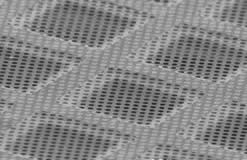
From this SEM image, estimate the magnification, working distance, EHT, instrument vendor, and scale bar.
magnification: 1.1 K X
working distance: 21 mm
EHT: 5 kV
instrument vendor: JEOL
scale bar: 10000 nm